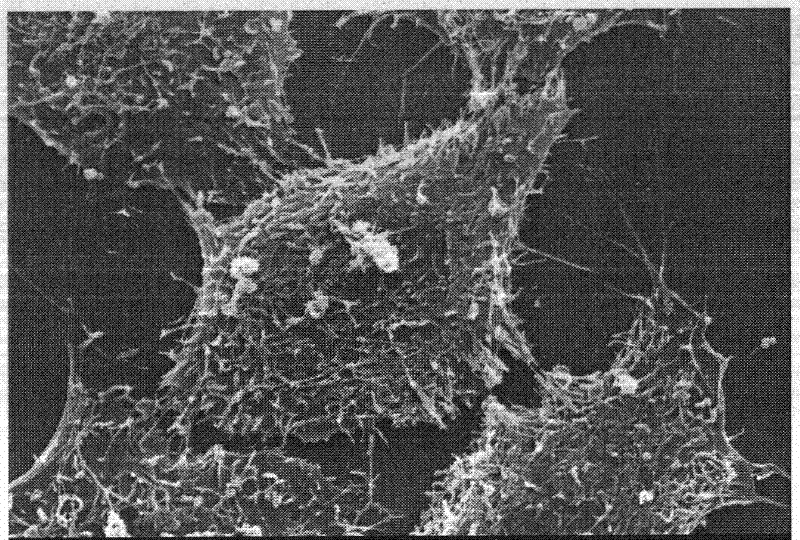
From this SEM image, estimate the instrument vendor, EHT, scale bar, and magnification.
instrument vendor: other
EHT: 25 kV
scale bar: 10000 nm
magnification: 3 K X